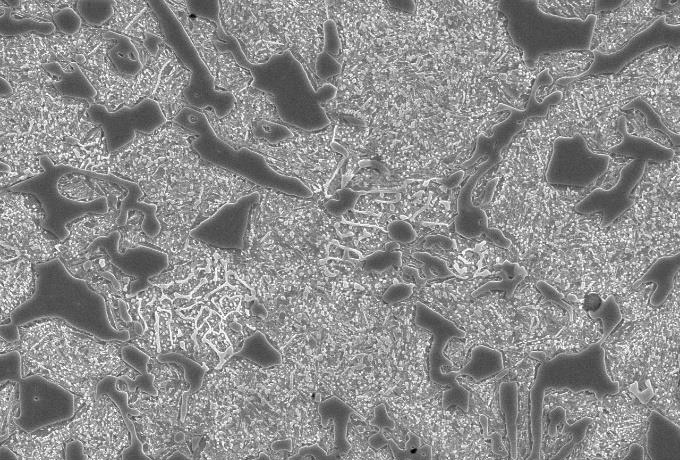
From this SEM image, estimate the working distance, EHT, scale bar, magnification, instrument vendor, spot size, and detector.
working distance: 12 mm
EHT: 20 kV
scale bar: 10000 nm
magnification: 1.5 K X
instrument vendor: JEOL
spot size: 50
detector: SEI